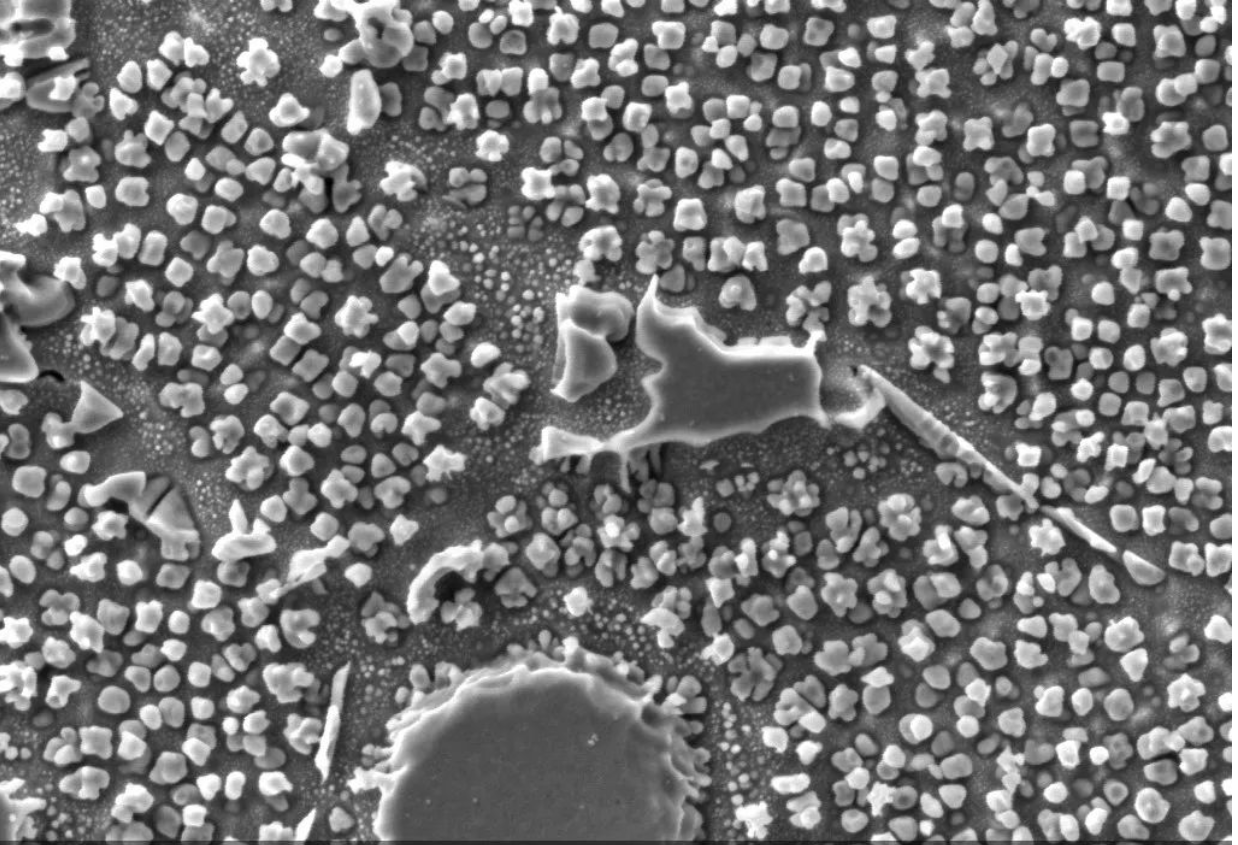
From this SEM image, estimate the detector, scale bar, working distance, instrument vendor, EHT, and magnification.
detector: SE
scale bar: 2000 nm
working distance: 7.253 mm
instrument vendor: other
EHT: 5 kV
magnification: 20 K X